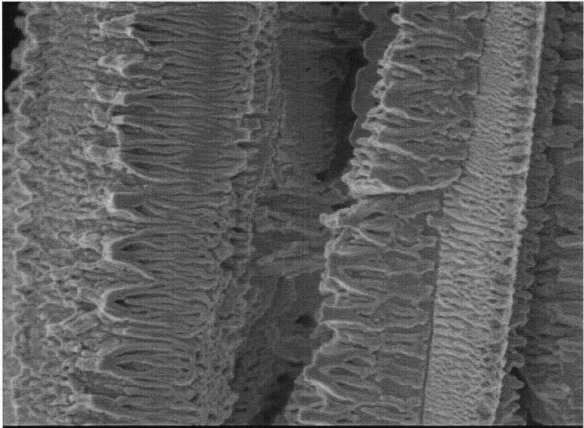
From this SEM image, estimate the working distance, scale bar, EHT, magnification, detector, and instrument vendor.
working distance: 3 mm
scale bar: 100 nm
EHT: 1 kV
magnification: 50 K X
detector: SEI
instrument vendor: JEOL